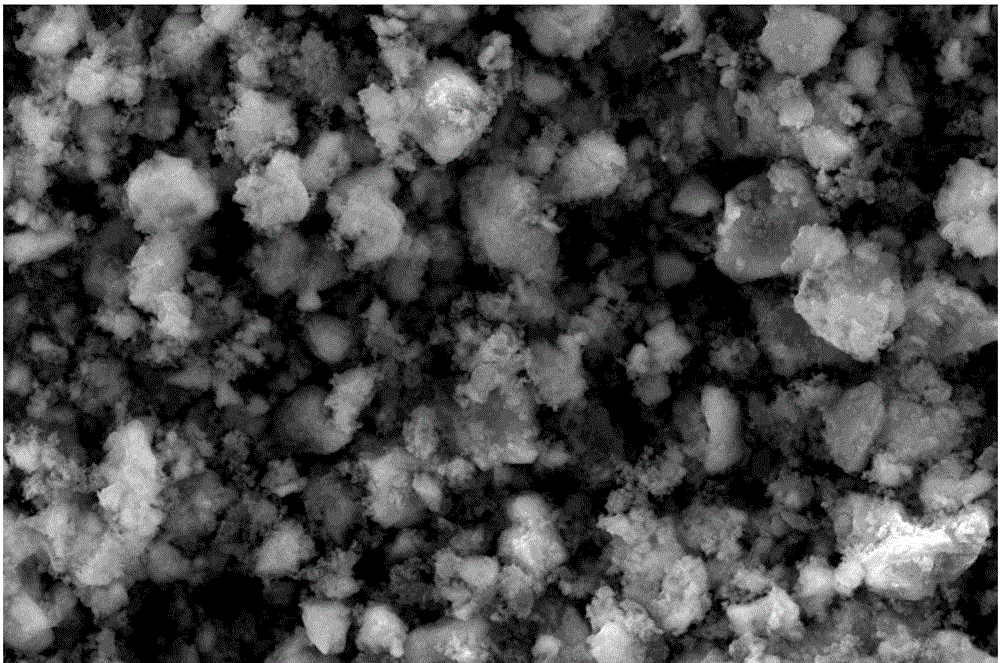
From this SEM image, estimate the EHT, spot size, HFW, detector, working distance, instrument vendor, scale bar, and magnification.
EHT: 20 kV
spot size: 3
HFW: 41.4 µm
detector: SE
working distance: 9.1 mm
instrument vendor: FEI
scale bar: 10000 nm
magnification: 10 K X